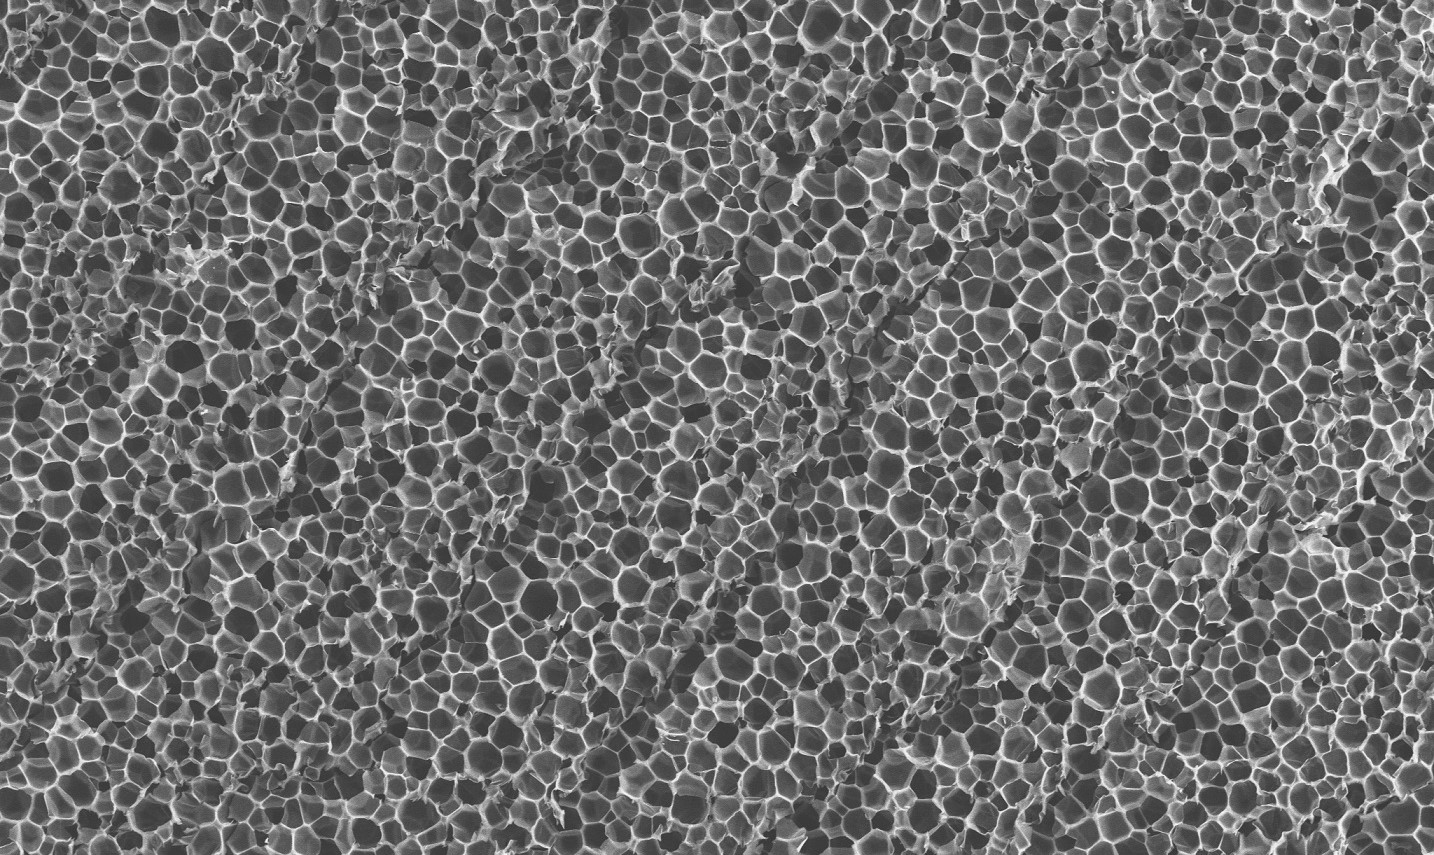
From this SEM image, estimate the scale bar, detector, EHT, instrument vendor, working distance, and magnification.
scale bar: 100000 nm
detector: SE2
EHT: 15 kV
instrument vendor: Zeiss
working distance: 7.3 mm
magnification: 0.1 K X